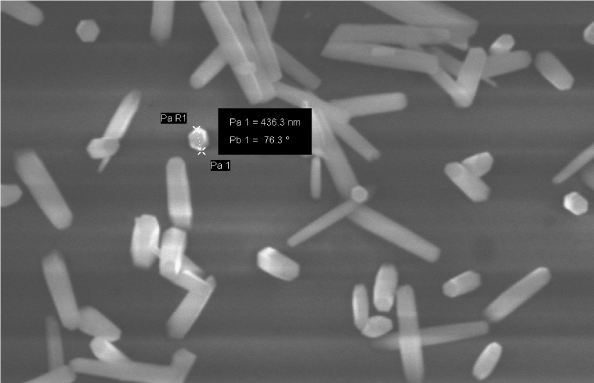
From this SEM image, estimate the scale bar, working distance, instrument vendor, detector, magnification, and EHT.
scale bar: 1000 nm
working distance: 7 mm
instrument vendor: Zeiss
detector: VPSE G3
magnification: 10 K X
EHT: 20 kV